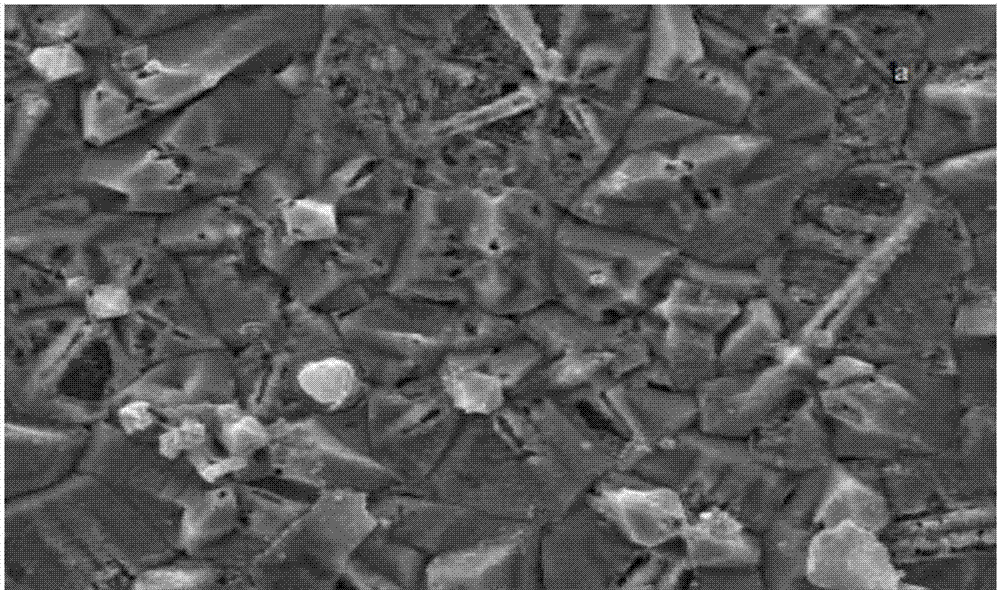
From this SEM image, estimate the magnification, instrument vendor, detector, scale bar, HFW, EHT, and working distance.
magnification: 5 K X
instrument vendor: Tescan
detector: SE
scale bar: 10000 nm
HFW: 55.4 µm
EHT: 20 kV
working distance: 6.23 mm